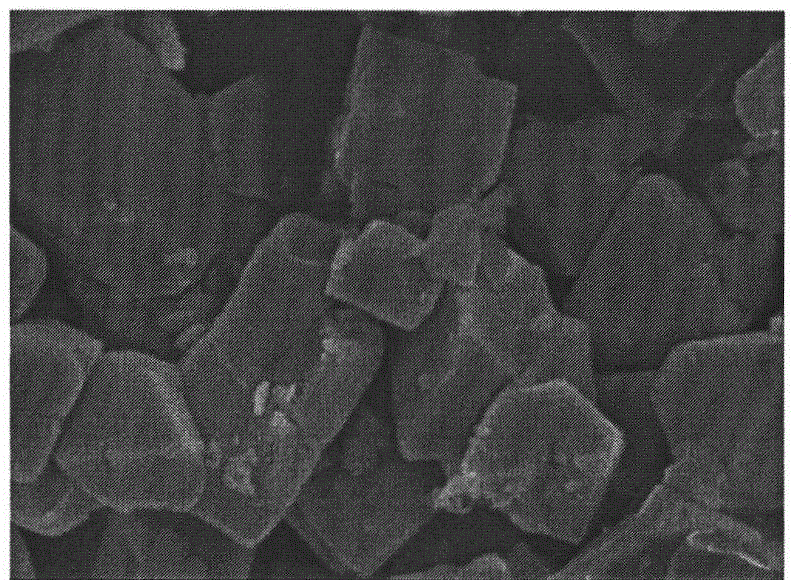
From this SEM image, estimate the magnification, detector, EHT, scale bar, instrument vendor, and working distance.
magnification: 10 K X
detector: SEI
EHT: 10 kV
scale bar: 1000 nm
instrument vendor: JEOL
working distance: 8.7 mm